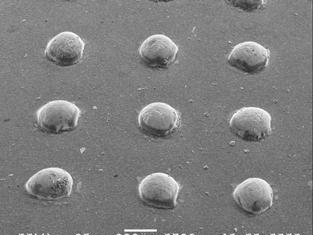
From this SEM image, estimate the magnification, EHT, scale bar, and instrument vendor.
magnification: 0.03 K X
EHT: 20 kV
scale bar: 333000 nm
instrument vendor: JEOL